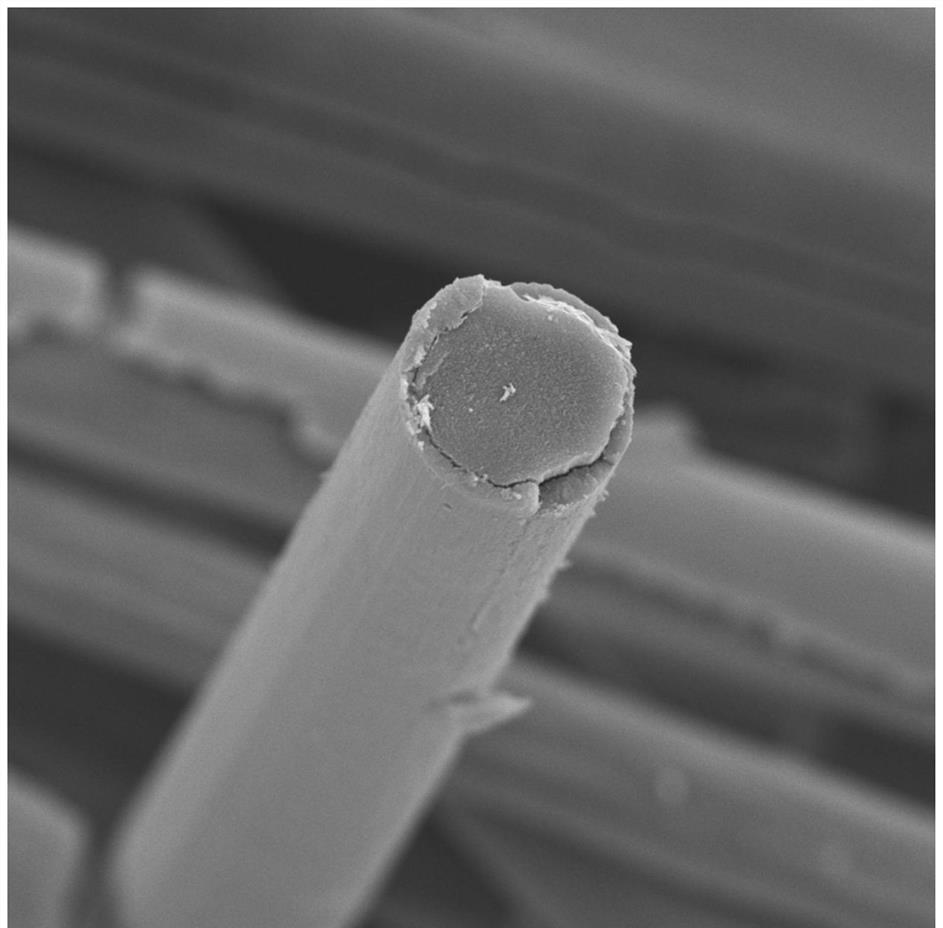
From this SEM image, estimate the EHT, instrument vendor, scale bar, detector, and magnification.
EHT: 10 kV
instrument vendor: Thermo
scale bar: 8000 nm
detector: SED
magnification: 9.5 K X